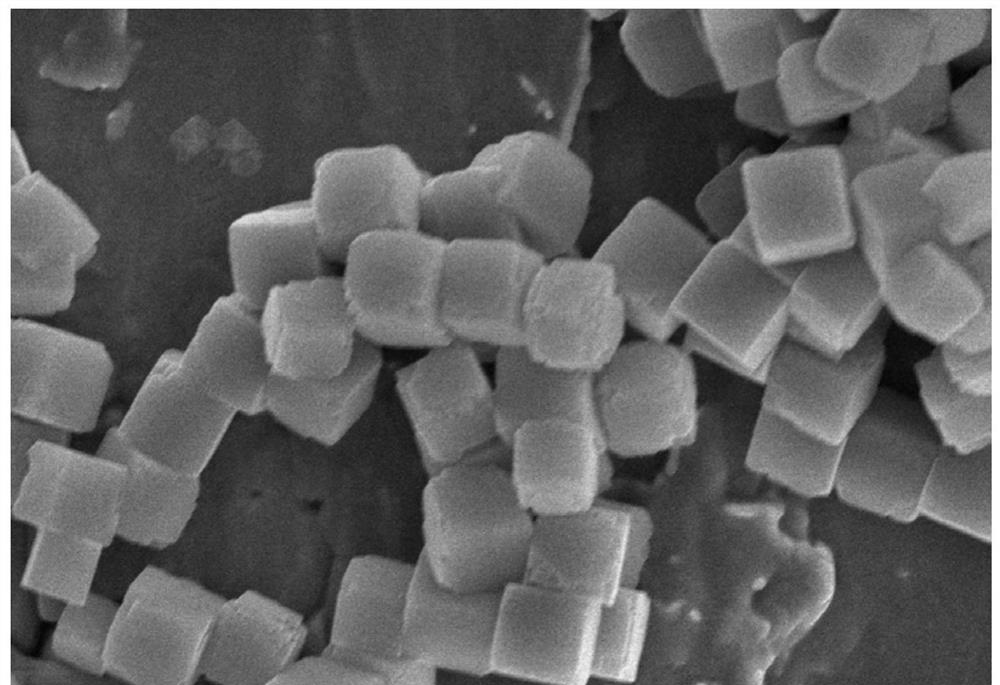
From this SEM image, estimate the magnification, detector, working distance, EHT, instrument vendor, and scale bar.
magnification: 22 K X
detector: SE(UL)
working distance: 10.8 mm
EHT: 8 kV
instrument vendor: Hitachi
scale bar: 2000 nm